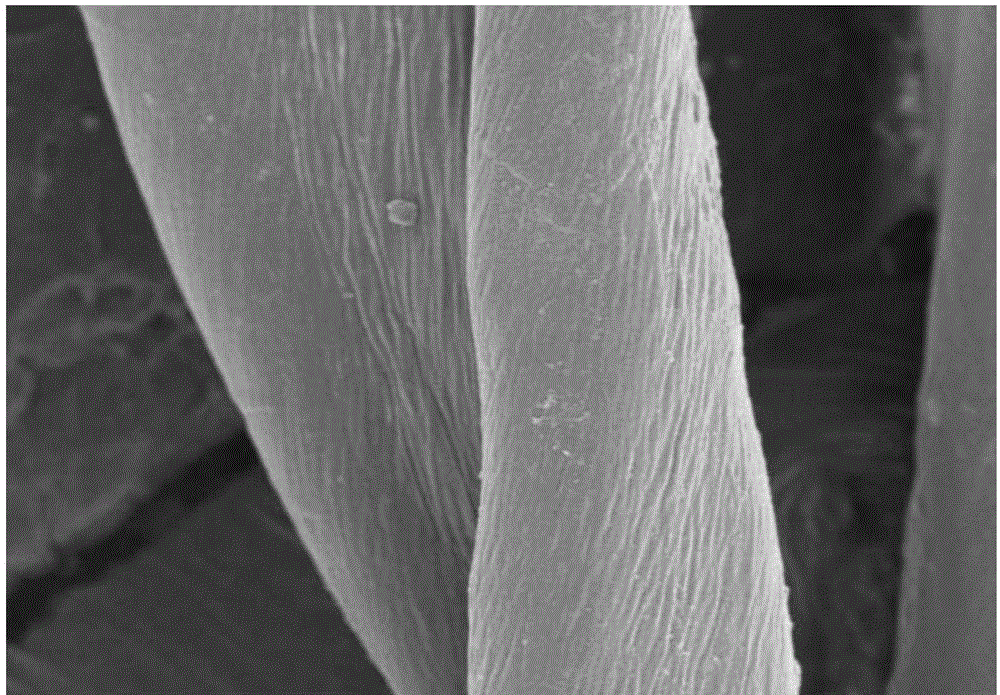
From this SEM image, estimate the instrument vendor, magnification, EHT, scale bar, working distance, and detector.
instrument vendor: Hitachi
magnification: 5 K X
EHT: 3 kV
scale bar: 10000 nm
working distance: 9.8 mm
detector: SE(M)